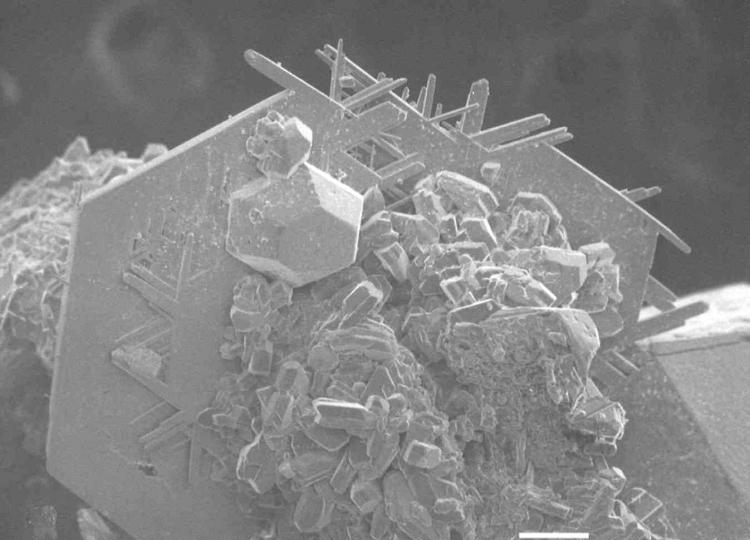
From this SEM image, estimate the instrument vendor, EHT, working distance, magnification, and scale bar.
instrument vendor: JEOL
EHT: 15 kV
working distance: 39 mm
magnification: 0.1 K X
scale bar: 100000 nm